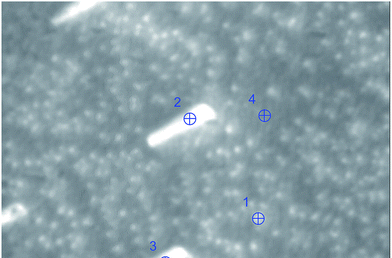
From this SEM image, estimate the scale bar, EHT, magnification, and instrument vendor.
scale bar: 500 nm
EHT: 20 kV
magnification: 40 K X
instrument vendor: other